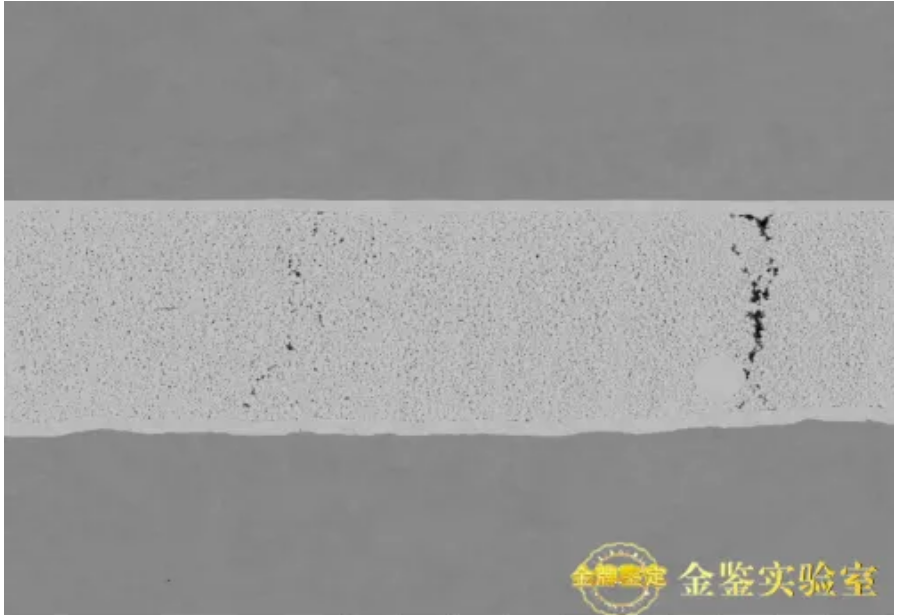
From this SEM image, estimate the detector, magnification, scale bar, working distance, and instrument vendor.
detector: NL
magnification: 1 K X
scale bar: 100000 nm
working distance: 7.5 mm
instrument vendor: Hitachi